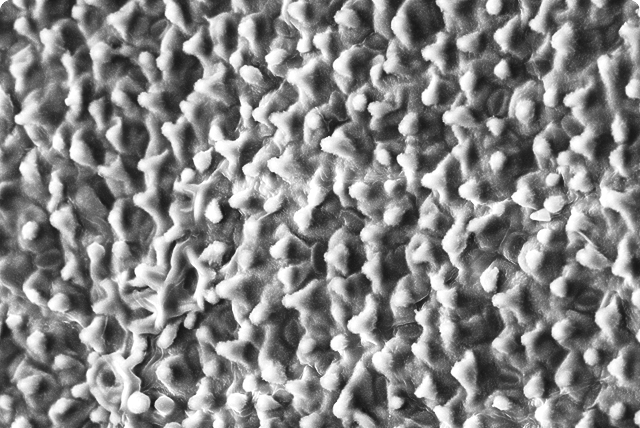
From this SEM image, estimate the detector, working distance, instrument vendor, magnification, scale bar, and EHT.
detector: SE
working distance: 12.1 mm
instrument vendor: Hitachi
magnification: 0.5 K X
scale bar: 100000 nm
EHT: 20 kV